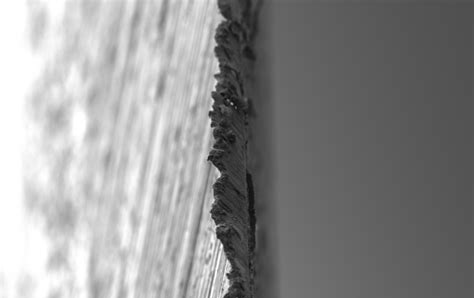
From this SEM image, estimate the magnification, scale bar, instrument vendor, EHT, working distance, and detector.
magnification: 0.5 K X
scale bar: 20000 nm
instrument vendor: Zeiss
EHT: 5 kV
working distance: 6.5 mm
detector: SE2